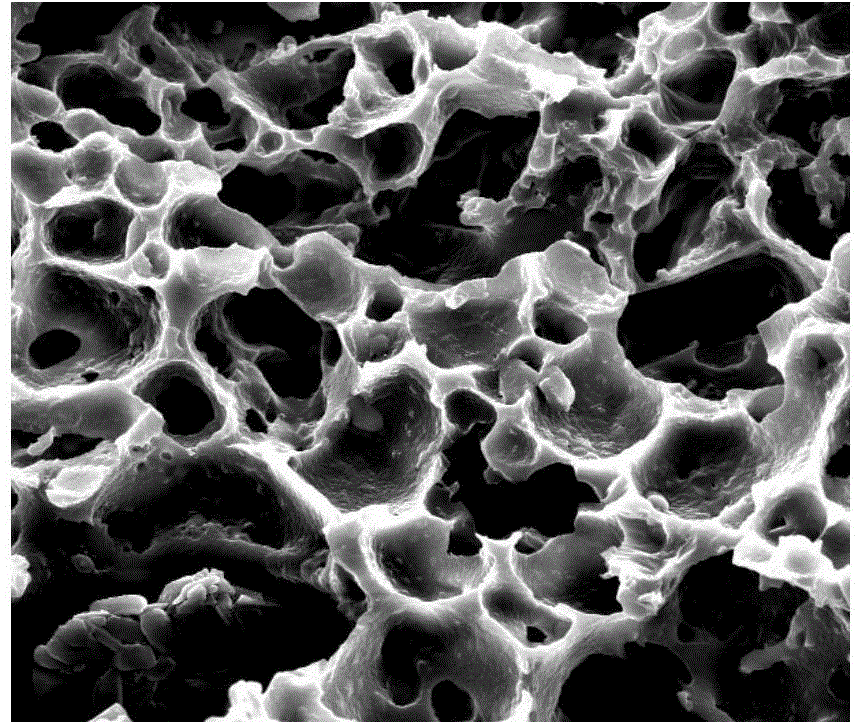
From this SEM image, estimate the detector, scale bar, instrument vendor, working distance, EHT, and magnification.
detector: SE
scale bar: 50000 nm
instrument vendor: FEI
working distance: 10.8 mm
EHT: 20 kV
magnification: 2 K X